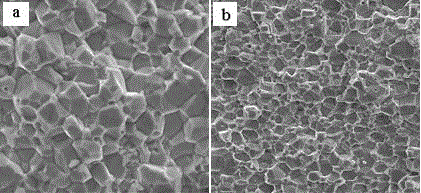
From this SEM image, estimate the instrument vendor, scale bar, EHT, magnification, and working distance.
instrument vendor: JEOL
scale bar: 20000 nm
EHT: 20 kV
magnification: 2 K X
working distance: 16.4 mm